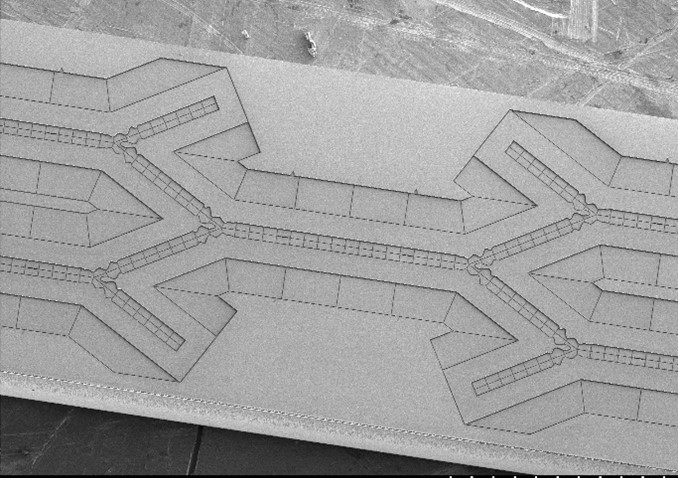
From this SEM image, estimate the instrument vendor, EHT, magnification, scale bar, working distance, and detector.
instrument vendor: Hitachi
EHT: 3 kV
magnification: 0.04 K X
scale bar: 1000000 nm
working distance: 8.1 mm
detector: SE(M)